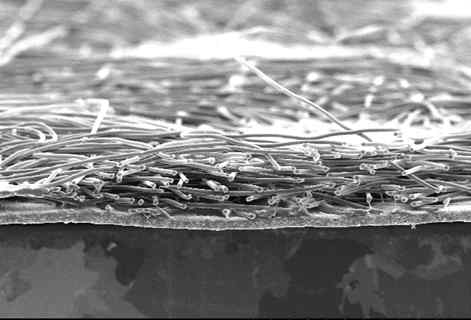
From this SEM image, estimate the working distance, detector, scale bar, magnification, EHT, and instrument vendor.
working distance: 23.6 mm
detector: SE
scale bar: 500000 nm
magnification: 0.1 K X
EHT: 20 kV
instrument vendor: JEOL